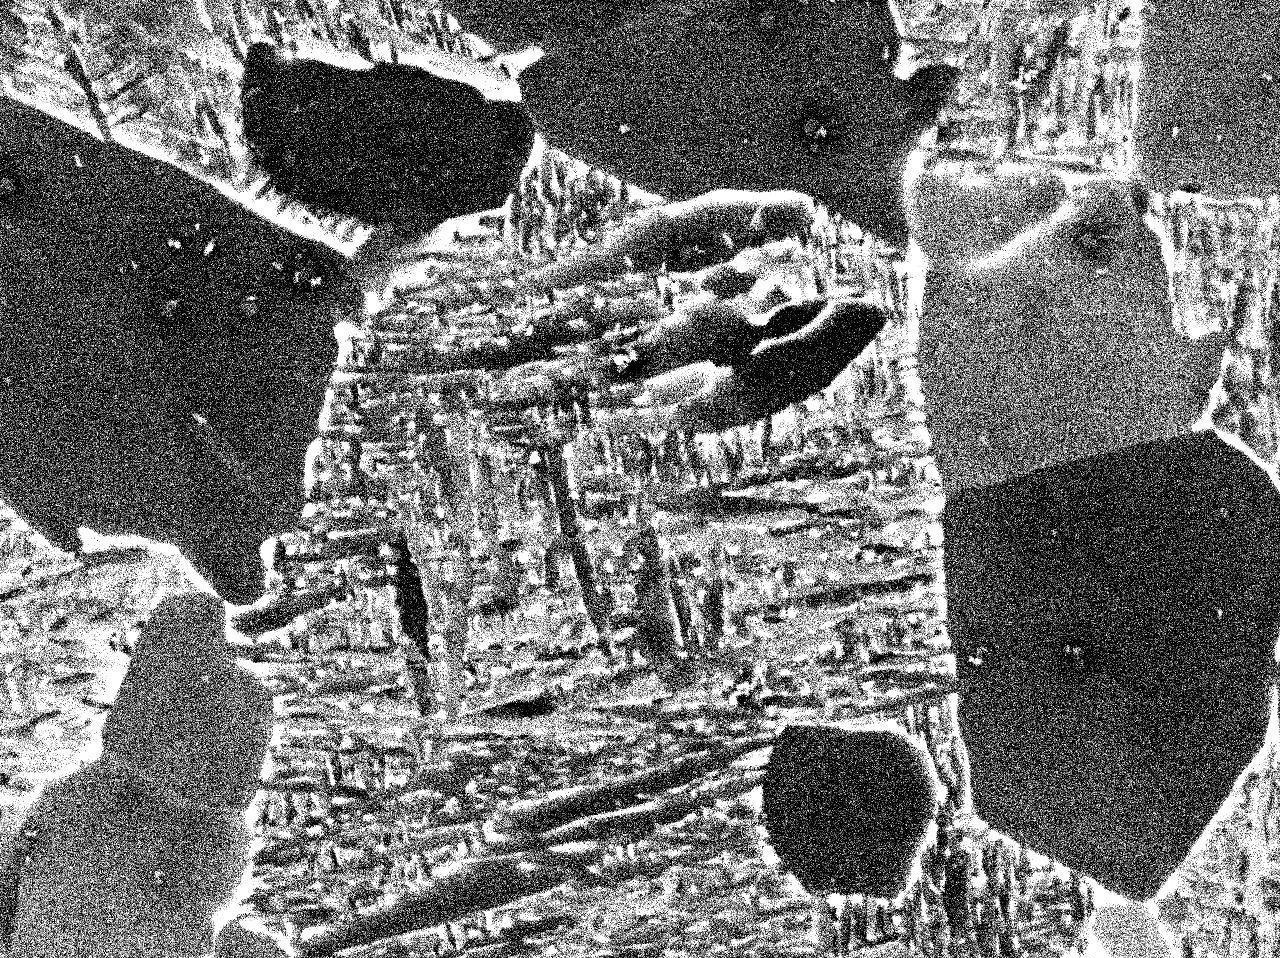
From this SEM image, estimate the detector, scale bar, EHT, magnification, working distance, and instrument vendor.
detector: COMPO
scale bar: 1000 nm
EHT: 15 kV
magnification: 4.5 K X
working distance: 10 mm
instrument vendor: JEOL